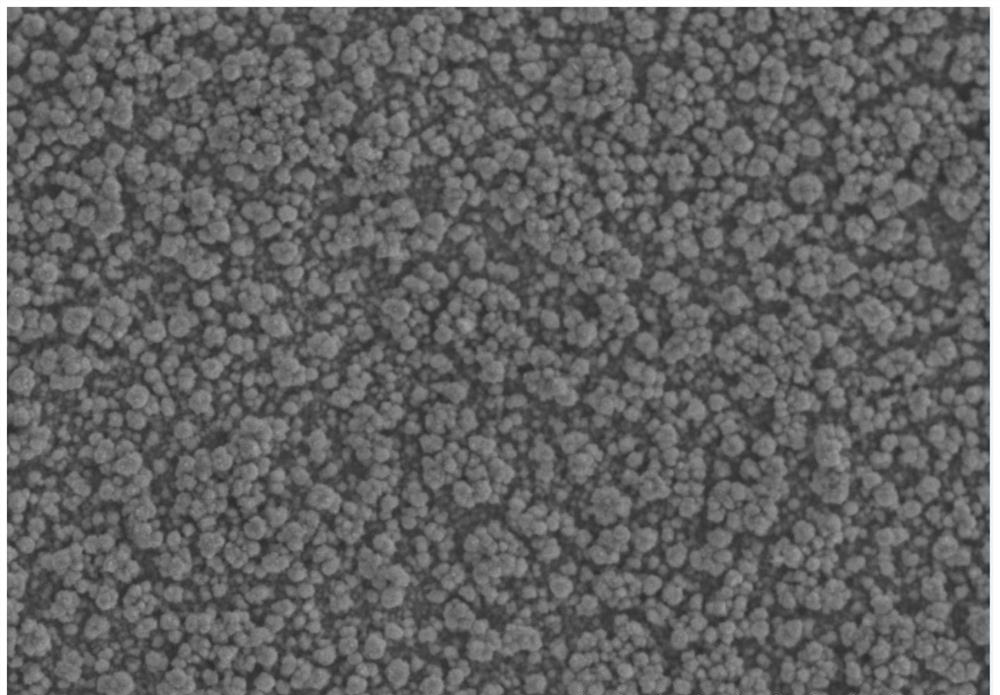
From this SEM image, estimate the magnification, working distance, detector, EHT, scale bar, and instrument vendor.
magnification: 1 K X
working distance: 9.2 mm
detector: SE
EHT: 25 kV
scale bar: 50000 nm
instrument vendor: Hitachi